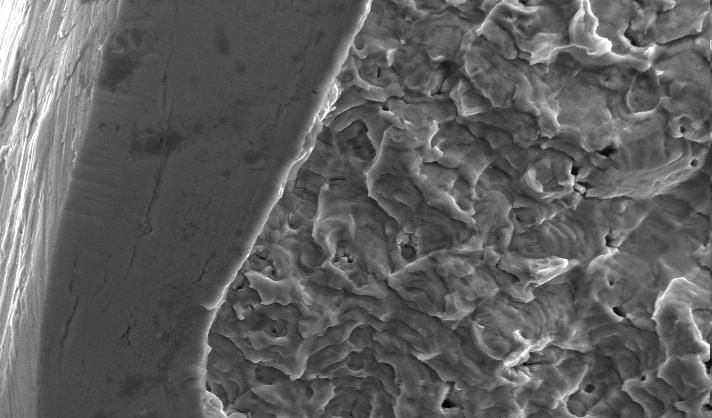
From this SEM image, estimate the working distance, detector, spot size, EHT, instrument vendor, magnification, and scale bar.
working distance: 10.1 mm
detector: SE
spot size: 4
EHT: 20 kV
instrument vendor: FEI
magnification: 3 K X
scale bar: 20000 nm